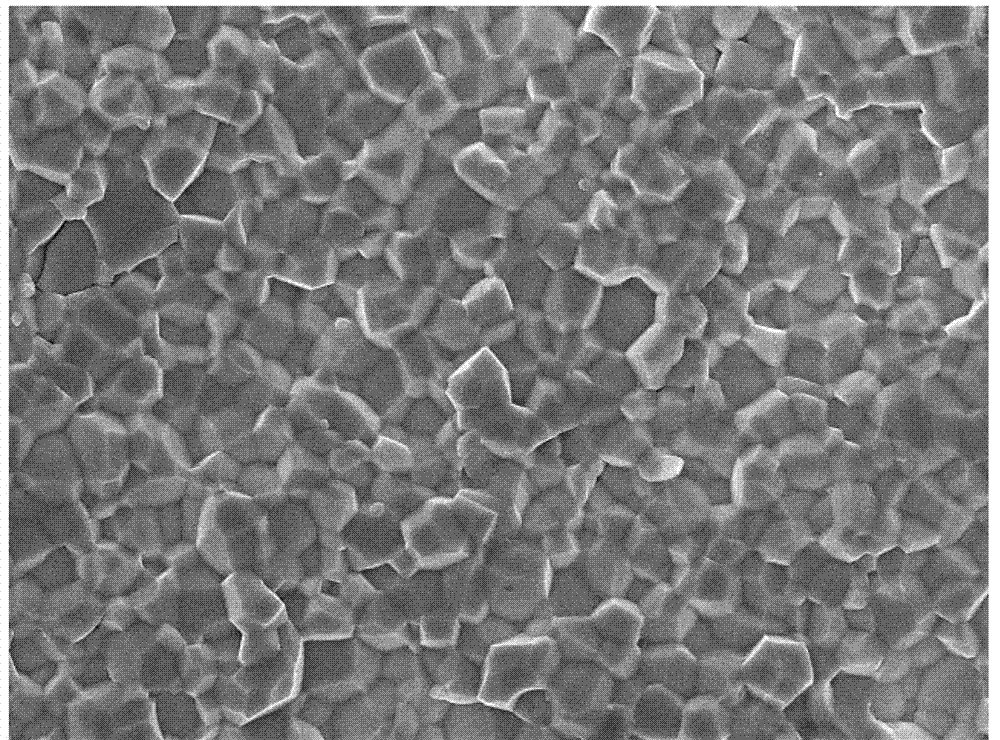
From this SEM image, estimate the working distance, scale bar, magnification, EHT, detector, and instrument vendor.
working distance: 6 mm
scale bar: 1000 nm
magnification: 5 K X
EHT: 10 kV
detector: SEI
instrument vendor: JEOL